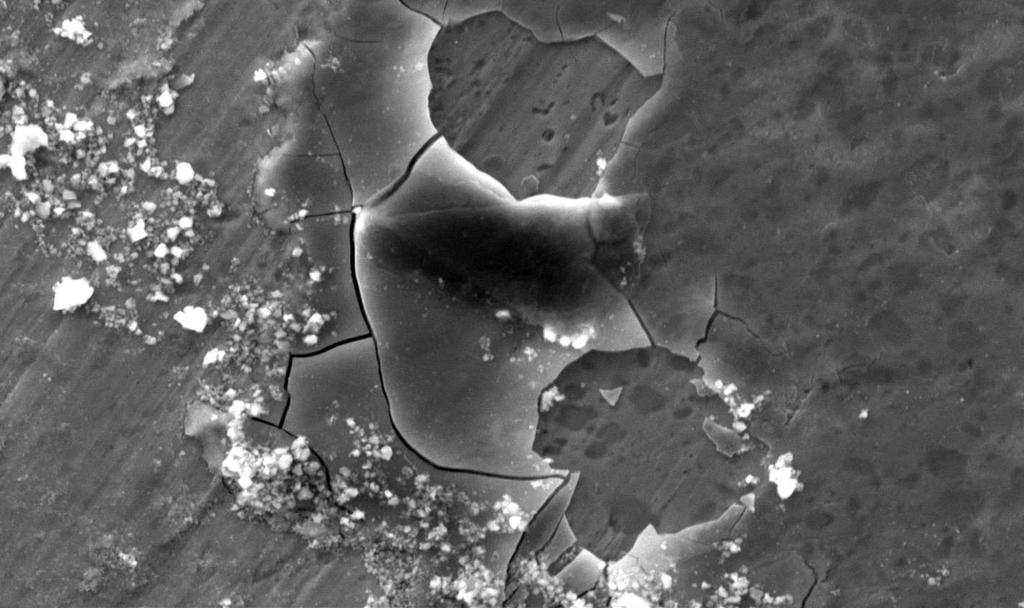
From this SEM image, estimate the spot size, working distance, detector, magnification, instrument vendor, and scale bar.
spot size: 5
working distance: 11.1 mm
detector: SE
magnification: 1.2 K X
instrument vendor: FEI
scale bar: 20000 nm